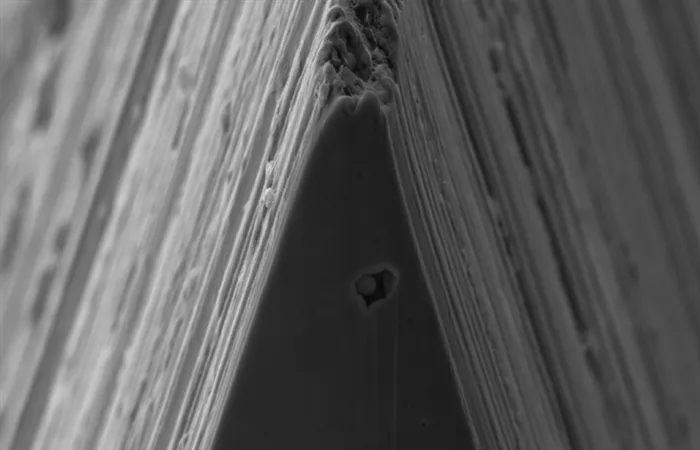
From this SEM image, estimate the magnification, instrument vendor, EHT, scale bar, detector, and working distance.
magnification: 10 K X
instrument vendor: Zeiss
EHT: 5 kV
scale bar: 1000 nm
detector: SE2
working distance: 6.5 mm